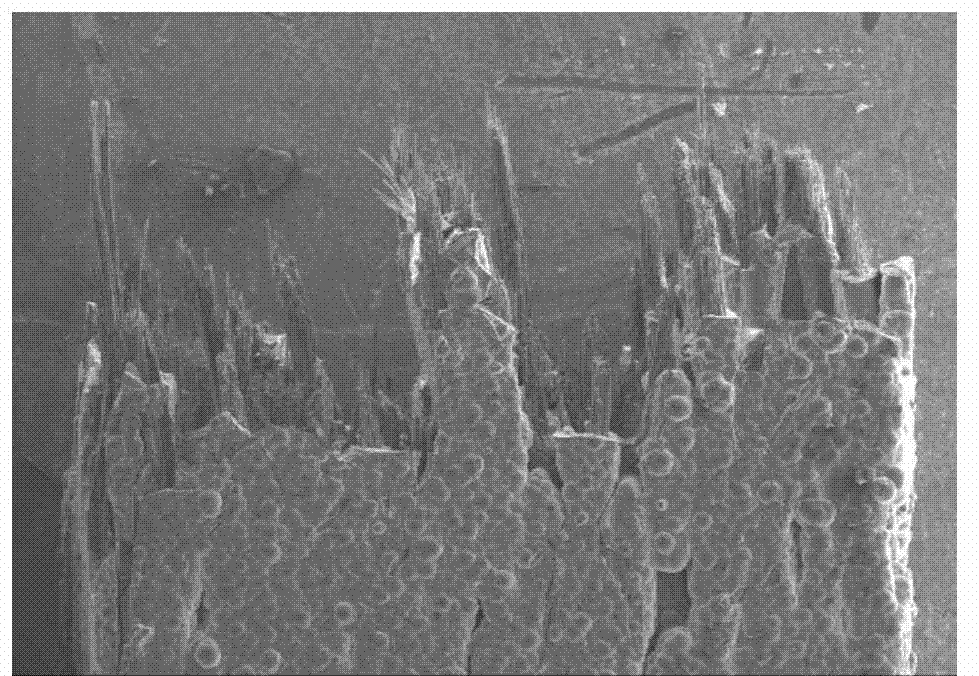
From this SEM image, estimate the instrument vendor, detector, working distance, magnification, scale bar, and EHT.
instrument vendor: Hitachi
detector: SE(M)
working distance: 15.5 mm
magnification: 0.035 K X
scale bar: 1000000 nm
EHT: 15 kV